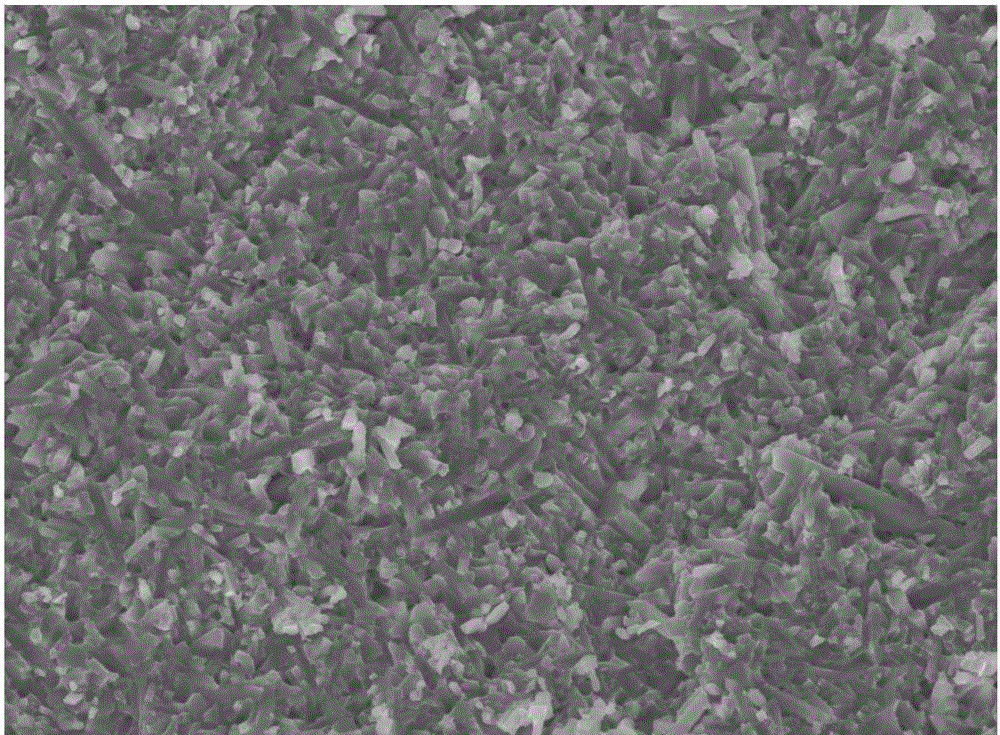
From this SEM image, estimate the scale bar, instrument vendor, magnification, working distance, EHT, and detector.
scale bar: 10000 nm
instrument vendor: JEOL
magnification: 2 K X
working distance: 7.8 mm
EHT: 15 kV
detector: SEI